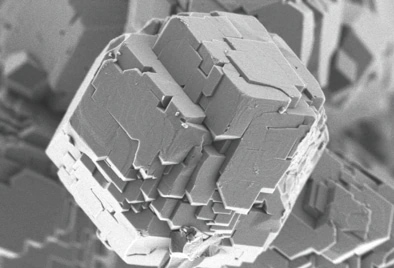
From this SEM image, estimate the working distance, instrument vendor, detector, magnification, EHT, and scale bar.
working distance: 2.1 mm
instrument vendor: Hitachi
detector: UD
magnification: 25 K X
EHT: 0.8 kV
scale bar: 2000 nm